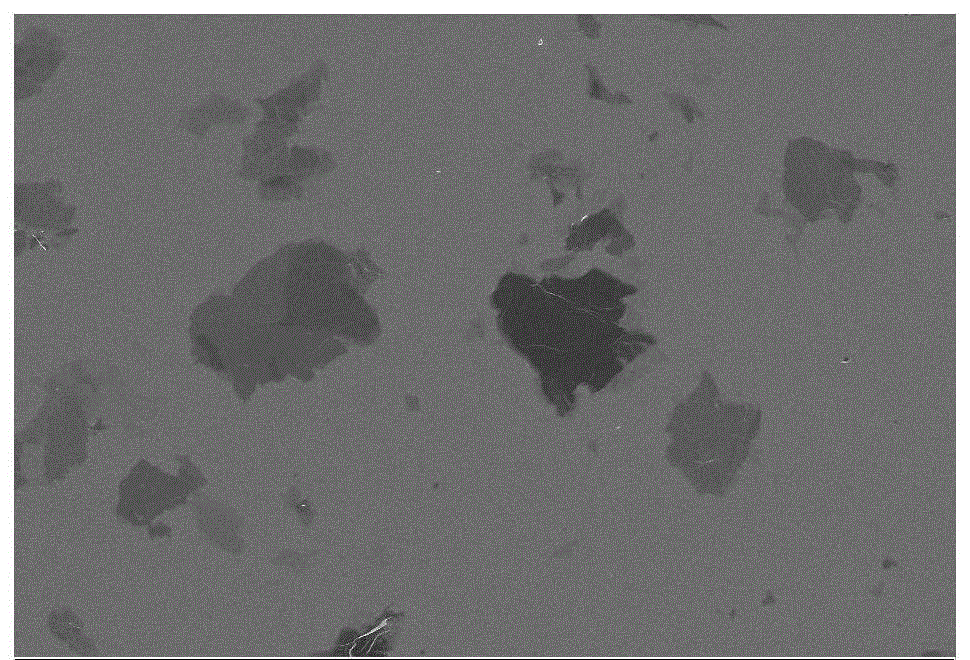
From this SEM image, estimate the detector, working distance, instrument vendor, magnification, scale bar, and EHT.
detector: InLens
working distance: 8.3 mm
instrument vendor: Zeiss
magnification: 0.5 K X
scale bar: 20000 nm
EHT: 5 kV